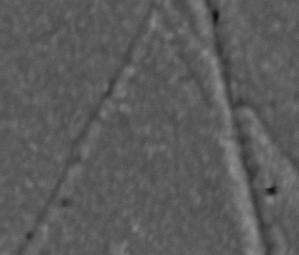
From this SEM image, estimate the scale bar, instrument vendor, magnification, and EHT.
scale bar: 500 nm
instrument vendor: JEOL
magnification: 20 K X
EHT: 10 kV